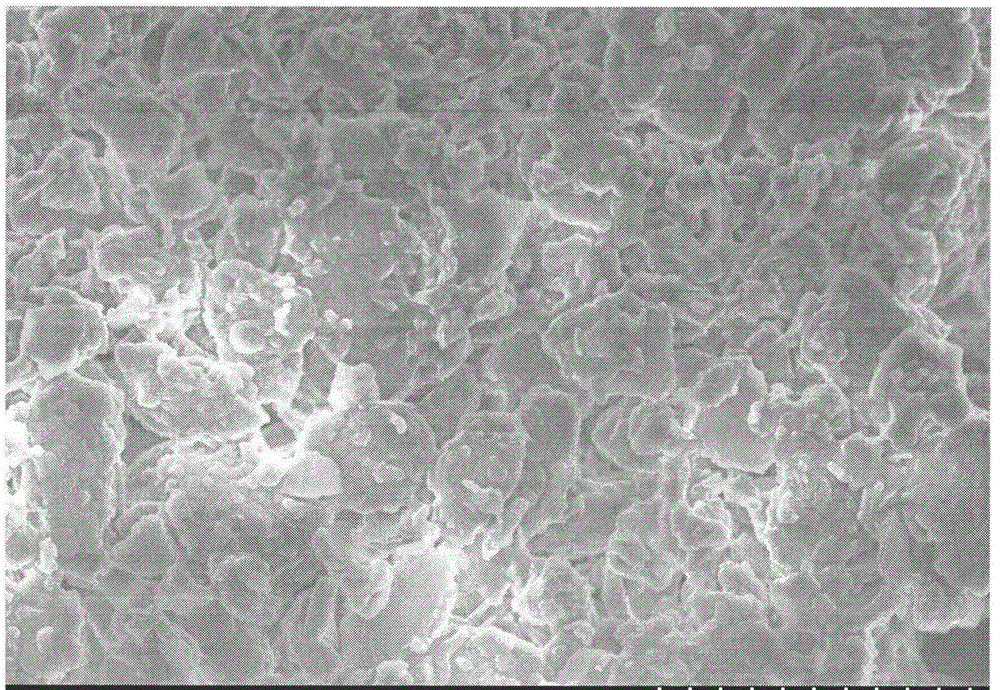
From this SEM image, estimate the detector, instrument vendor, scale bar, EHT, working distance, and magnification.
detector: SE(M)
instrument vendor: Hitachi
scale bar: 10000 nm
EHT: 5 kV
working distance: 7.7 mm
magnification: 4 K X